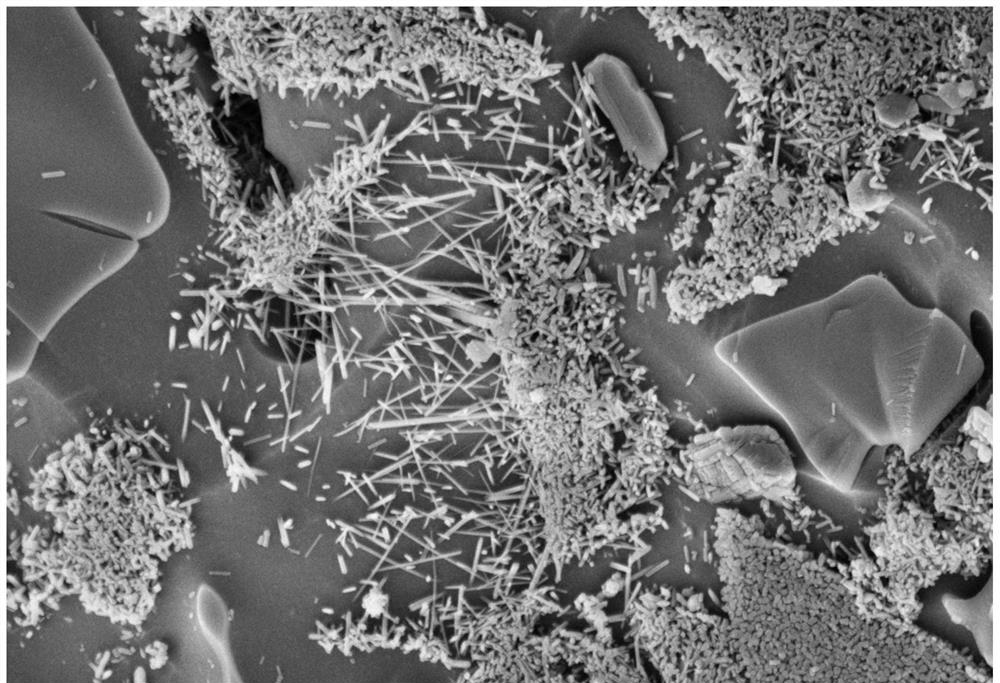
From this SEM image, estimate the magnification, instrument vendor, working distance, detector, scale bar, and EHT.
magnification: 10 K X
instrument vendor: Zeiss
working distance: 10 mm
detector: SE2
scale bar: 1000 nm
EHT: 5 kV